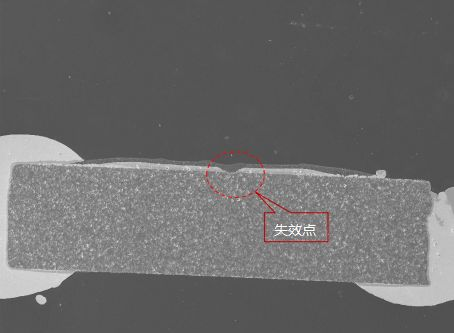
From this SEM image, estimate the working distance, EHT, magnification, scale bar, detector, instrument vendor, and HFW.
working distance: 15.04 mm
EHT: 20 kV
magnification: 0.16 K X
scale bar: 500000 nm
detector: SE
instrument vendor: Tescan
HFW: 1730 µm